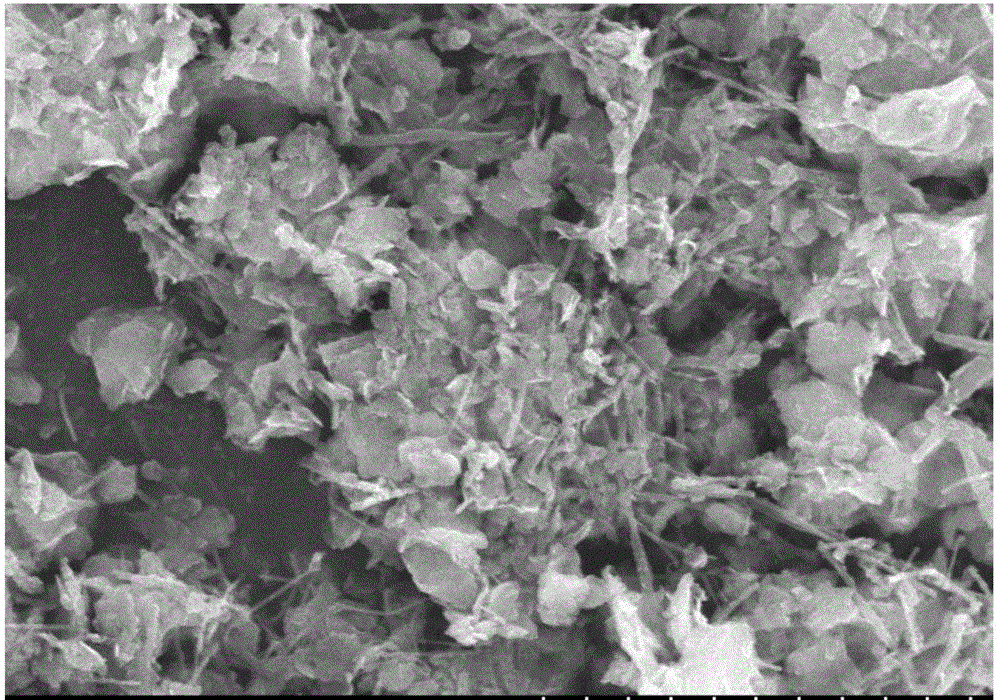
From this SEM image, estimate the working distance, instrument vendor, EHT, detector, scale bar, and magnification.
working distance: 9.3 mm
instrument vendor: Hitachi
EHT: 15 kV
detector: SE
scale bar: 10000 nm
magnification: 5.5 K X